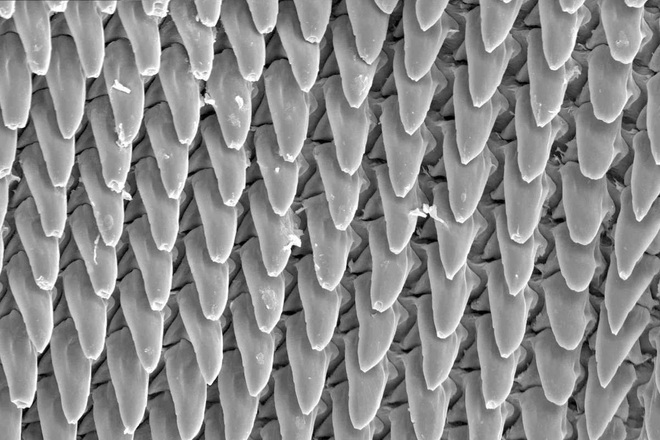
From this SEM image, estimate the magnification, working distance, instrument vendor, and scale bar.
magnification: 0.5 K X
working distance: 16.4 mm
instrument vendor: Hitachi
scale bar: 100000 nm